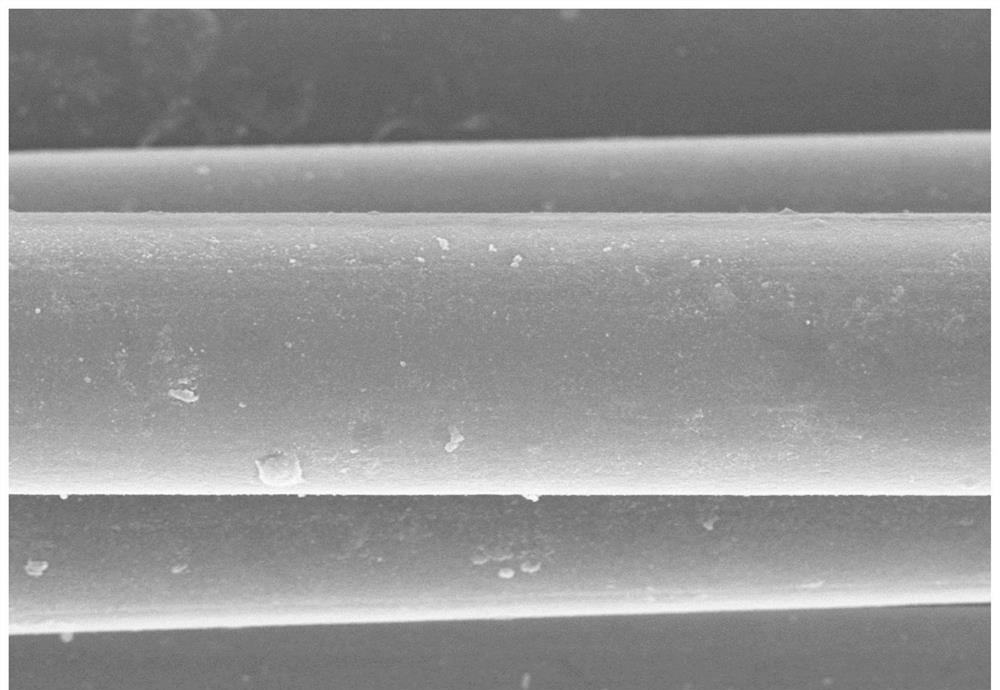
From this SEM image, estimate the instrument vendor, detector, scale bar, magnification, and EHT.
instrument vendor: Hitachi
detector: SE(M)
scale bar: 10000 nm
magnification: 5 K X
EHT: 8 kV